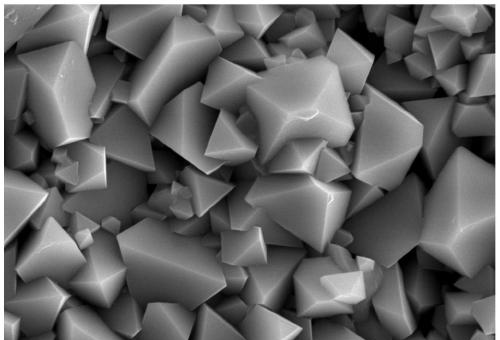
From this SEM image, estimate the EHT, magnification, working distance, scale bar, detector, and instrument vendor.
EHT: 10 kV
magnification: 20 K X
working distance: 11.1 mm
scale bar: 200 nm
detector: SE2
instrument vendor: Zeiss